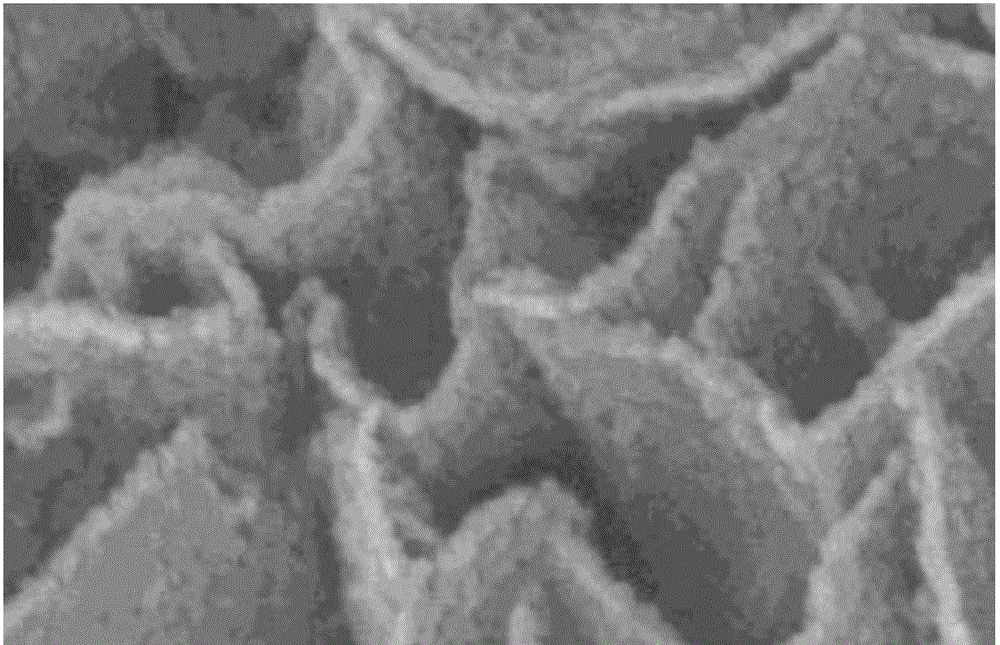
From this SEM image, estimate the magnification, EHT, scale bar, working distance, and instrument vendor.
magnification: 200 K X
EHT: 10 kV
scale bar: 200 nm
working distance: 13.6 mm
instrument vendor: Hitachi